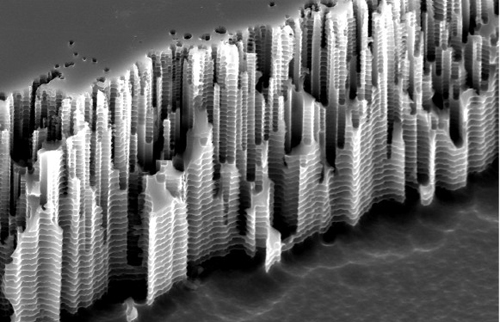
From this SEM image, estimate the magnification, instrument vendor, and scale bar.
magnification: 20 K X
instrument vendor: FEI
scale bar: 2000 nm